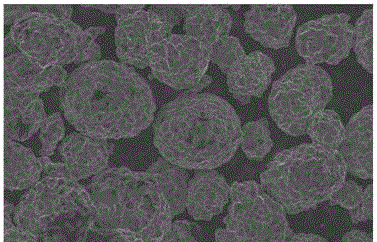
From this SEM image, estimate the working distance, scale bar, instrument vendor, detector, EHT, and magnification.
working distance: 7.8 mm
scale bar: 2000 nm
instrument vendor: Zeiss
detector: InLens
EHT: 5 kV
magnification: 2 K X